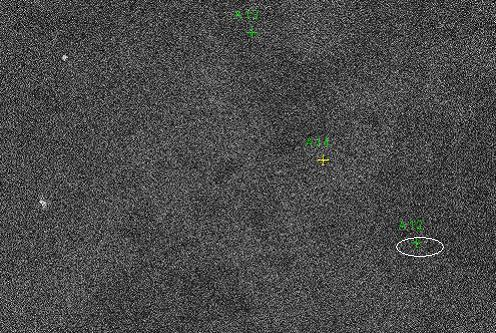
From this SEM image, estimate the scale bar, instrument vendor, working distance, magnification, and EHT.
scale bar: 70000 nm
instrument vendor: Tescan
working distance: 12.4 mm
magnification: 1 K X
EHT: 20 kV